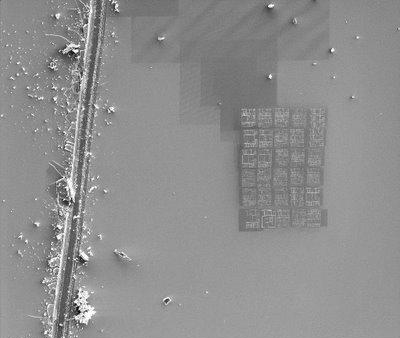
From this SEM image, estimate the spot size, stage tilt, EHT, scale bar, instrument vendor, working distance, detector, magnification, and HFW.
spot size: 3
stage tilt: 0°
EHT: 5 kV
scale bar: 50000 nm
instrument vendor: FEI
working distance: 5.01 mm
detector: SED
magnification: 0.5 K X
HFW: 304 µm